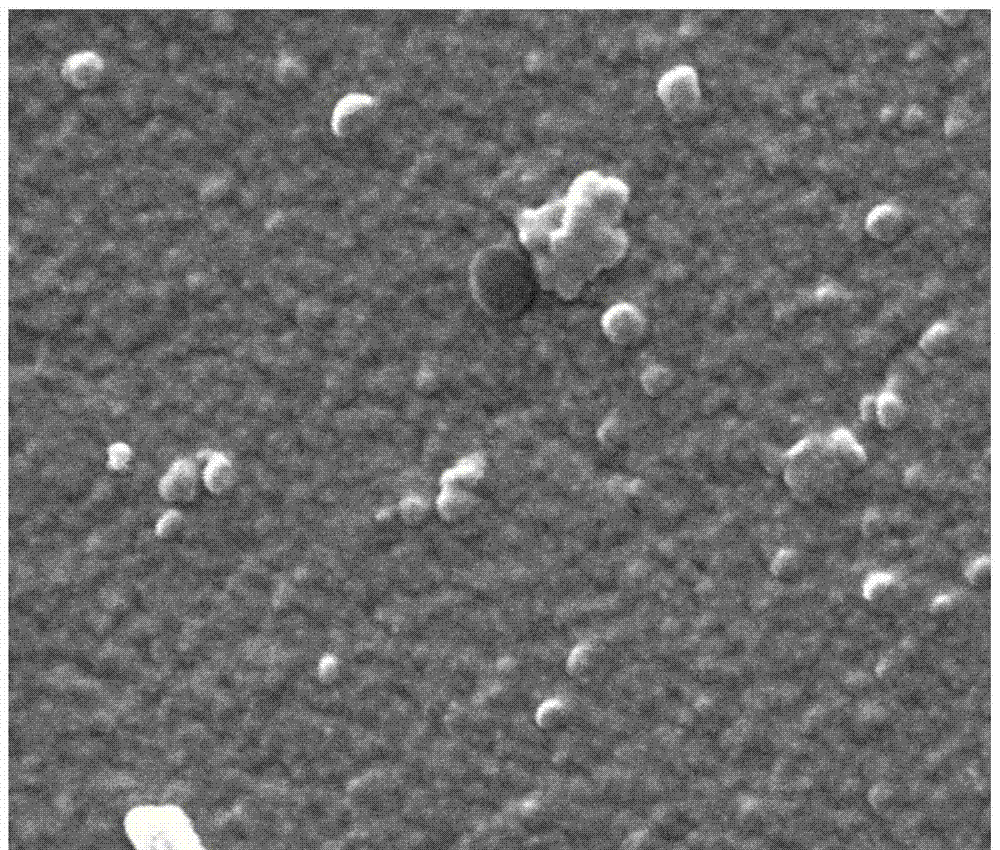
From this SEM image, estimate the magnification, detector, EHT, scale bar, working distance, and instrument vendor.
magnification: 100 K X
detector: SE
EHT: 30 kV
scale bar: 1000 nm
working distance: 10.6 mm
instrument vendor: FEI